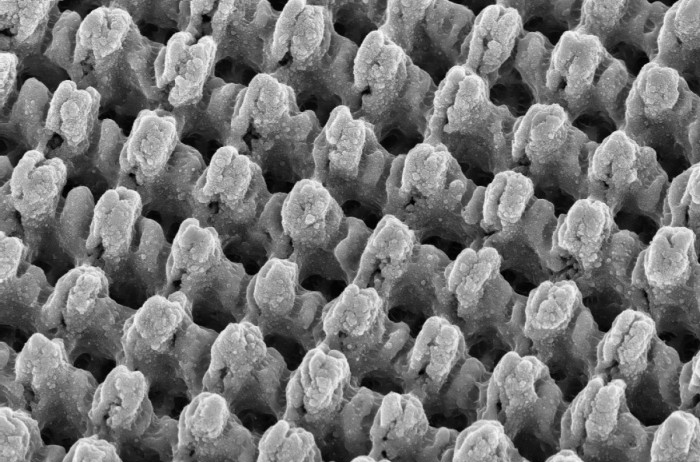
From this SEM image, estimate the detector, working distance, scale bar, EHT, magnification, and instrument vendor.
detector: InLens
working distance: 7.3 mm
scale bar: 2000 nm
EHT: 15 kV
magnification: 5 K X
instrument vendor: Zeiss